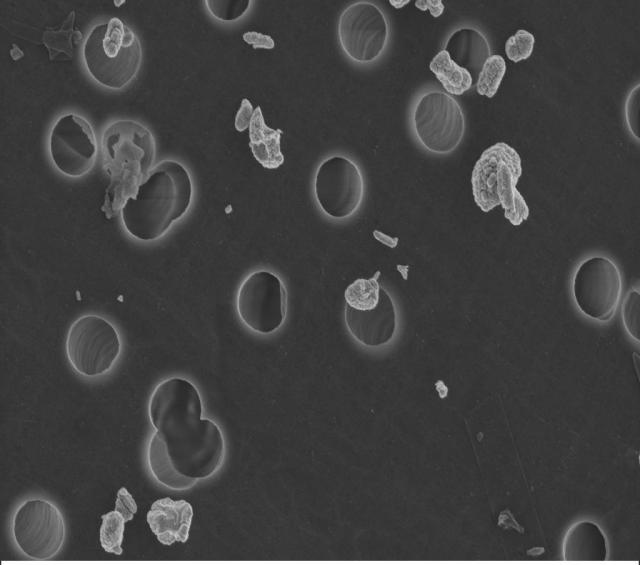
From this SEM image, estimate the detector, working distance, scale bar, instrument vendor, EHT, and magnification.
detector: InLens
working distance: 3 mm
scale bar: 1000 nm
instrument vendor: Zeiss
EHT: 3 kV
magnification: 30 K X